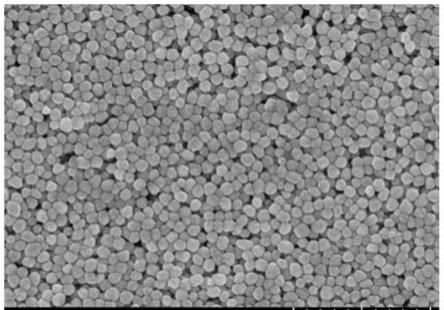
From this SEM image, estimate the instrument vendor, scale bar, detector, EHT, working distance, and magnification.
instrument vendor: Hitachi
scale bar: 1000 nm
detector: SE(UL)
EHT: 10 kV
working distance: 6.9 mm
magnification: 40 K X